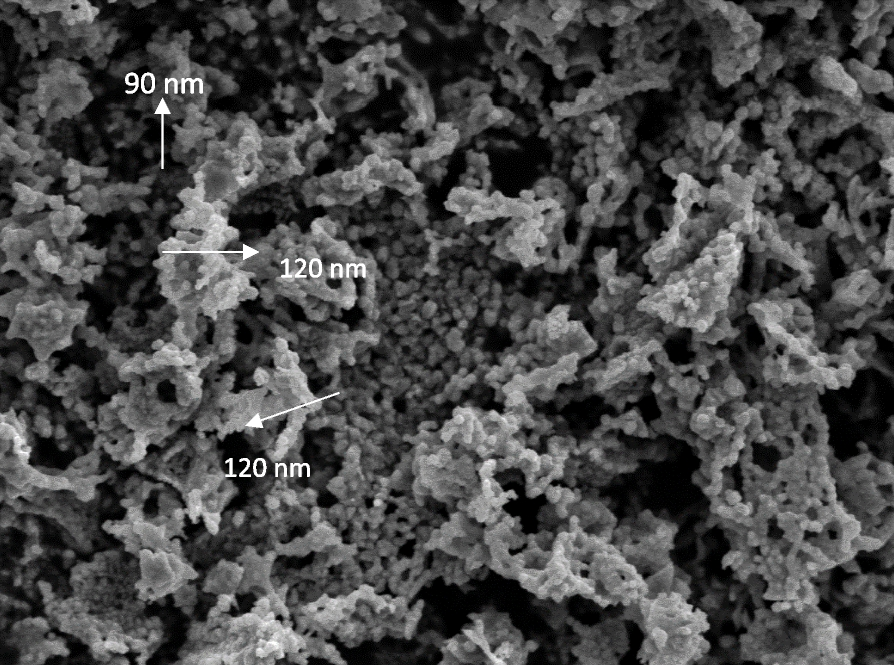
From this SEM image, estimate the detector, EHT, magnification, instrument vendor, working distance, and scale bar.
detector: LED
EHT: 6 kV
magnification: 10 K X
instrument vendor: JEOL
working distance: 9.6 mm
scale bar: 1000 nm